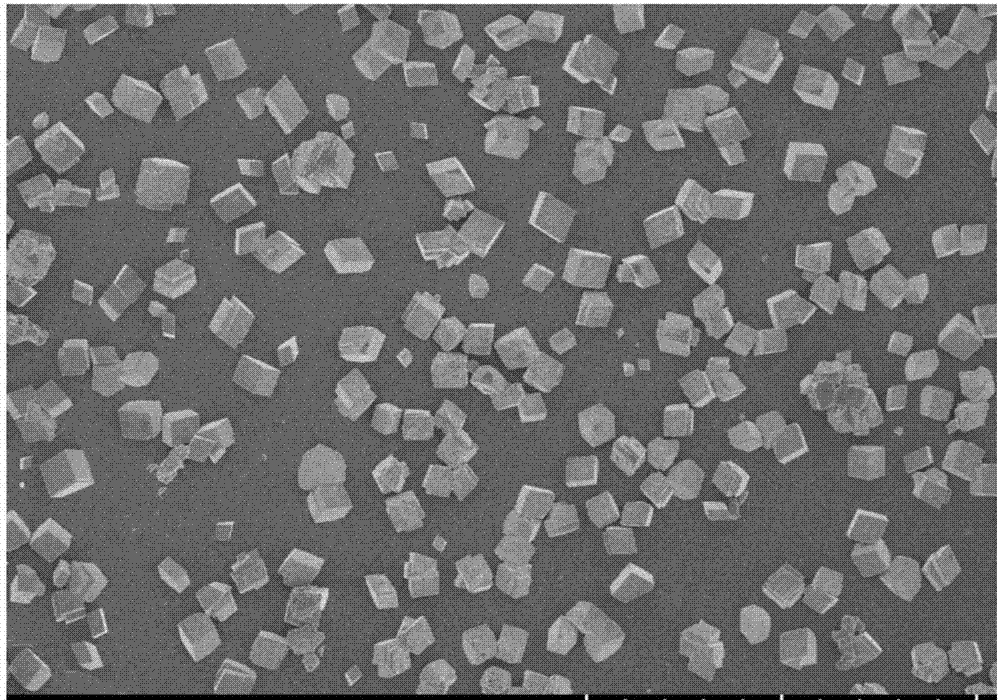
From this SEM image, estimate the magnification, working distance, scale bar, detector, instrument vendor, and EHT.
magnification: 0.5 K X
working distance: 8.7 mm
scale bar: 100000 nm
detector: SE(U)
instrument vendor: Hitachi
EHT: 5 kV